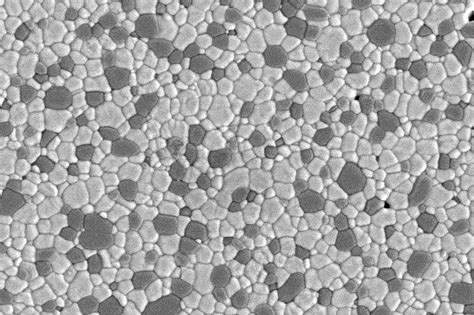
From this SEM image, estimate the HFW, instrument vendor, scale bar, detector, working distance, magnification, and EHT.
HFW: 11.43 µm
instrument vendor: Zeiss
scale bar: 2000 nm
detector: SE2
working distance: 8.6 mm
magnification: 10 K X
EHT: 5 kV